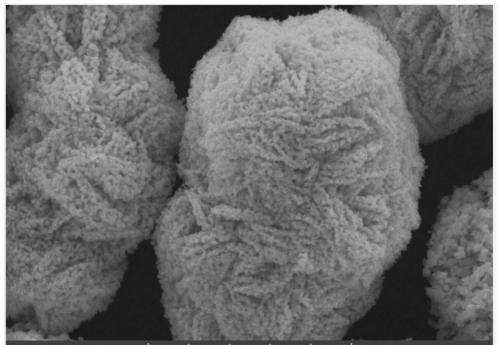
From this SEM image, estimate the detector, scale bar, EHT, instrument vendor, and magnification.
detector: SEM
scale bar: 10000 nm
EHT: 25 kV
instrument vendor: other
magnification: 5 K X